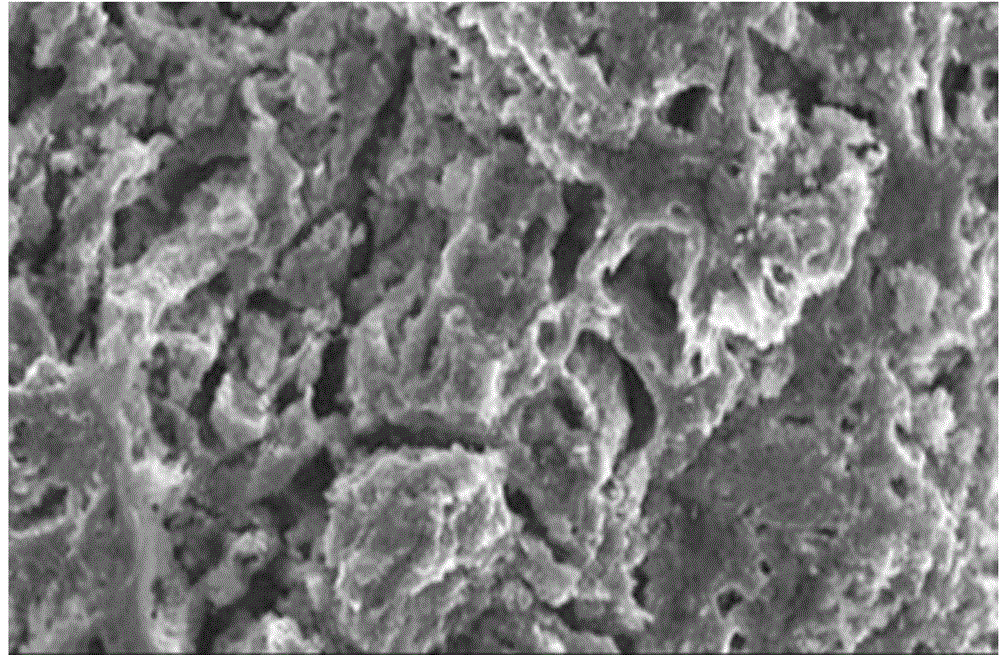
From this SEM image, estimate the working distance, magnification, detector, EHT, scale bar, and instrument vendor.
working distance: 9 mm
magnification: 5 K X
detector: SEI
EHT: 5 kV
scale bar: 1000 nm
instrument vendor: JEOL